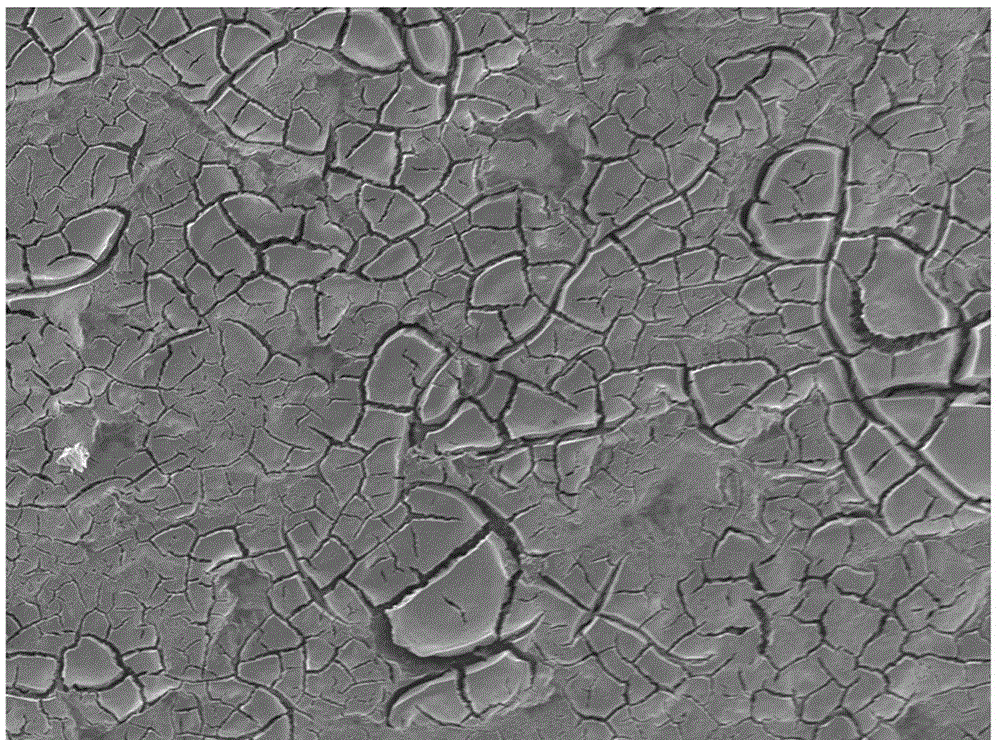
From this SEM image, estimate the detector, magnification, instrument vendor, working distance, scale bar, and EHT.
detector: LED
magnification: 0.5 K X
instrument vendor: JEOL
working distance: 9.7 mm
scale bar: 10000 nm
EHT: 5 kV